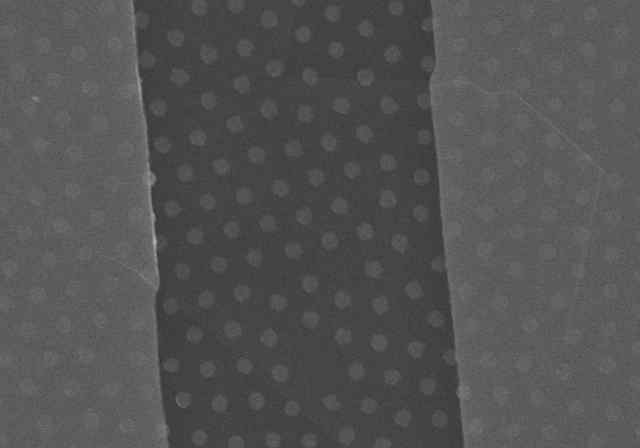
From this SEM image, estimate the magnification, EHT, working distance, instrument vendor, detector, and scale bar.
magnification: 7 K X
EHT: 15 kV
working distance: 15.1 mm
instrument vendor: Hitachi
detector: SE(U)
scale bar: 5000 nm